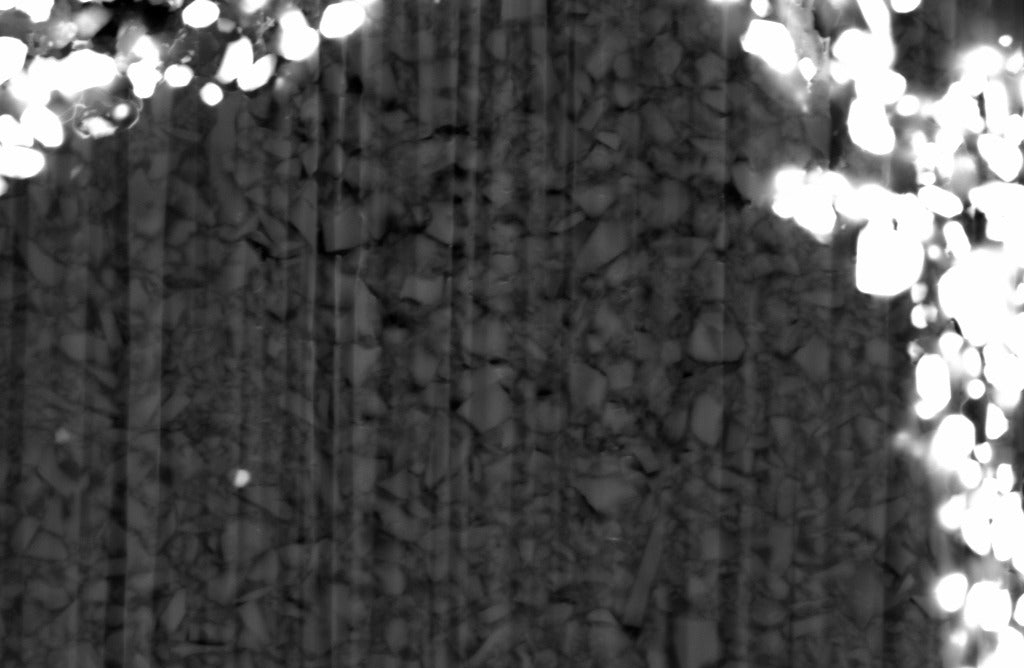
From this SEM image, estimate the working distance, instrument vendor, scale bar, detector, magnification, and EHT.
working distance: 9.2 mm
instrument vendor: Zeiss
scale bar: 1000 nm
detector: BSD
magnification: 10 K X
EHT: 10 kV